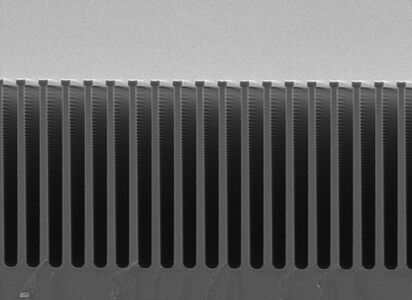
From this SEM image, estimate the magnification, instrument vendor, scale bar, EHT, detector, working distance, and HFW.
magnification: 3.5 K X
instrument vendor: JEOL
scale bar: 1000 nm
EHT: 3 kV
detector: SED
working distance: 8 mm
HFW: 36.6 µm